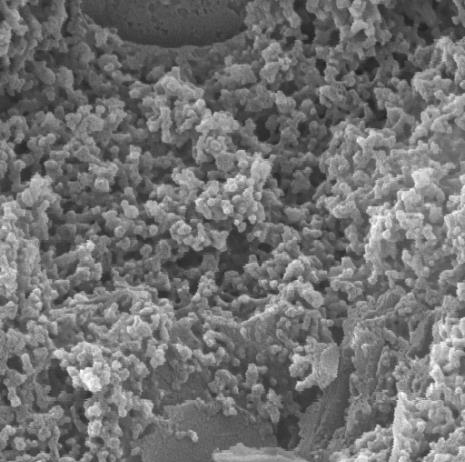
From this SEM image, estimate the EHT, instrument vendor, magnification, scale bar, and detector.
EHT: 20 kV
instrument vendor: Tescan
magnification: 50 K X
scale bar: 500 nm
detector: SE